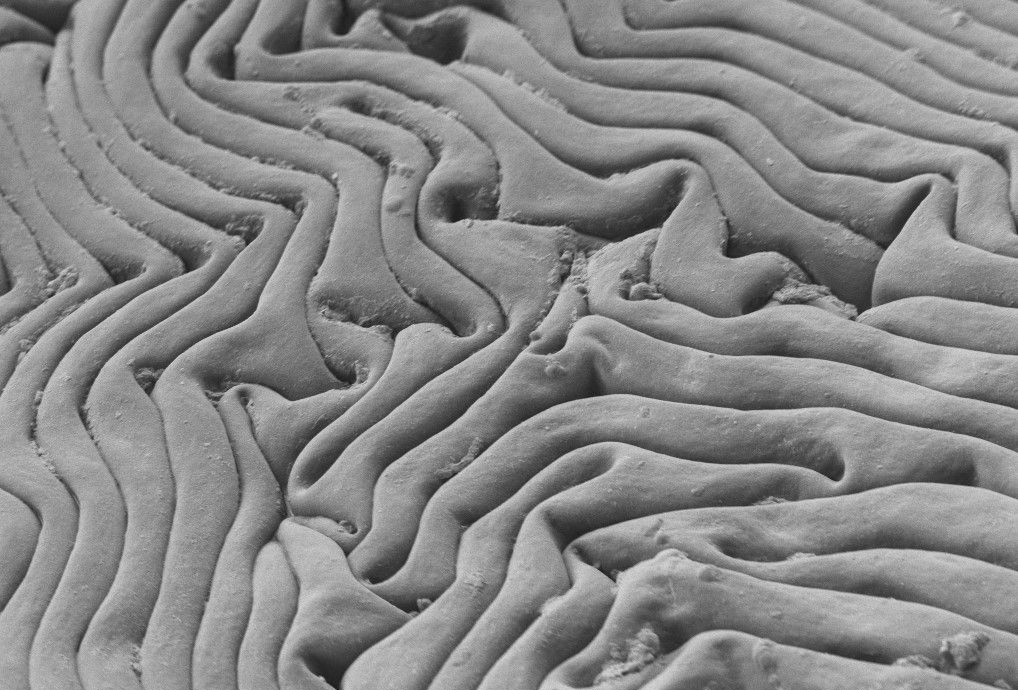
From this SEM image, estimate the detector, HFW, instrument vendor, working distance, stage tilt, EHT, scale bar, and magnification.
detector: SESI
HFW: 90.82 µm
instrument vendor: Zeiss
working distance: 4.7 mm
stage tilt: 0°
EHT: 0.9 kV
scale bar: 2000 nm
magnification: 3.33 K X